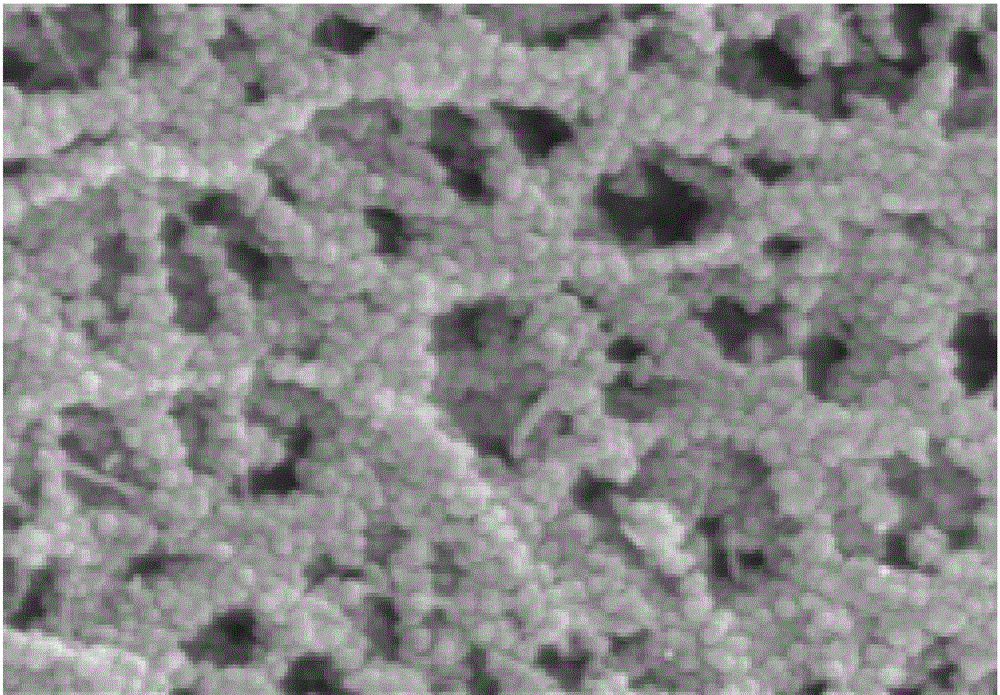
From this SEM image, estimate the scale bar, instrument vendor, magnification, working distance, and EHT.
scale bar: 500 nm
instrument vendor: Hitachi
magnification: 100 K X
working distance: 13.5 mm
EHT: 5 kV